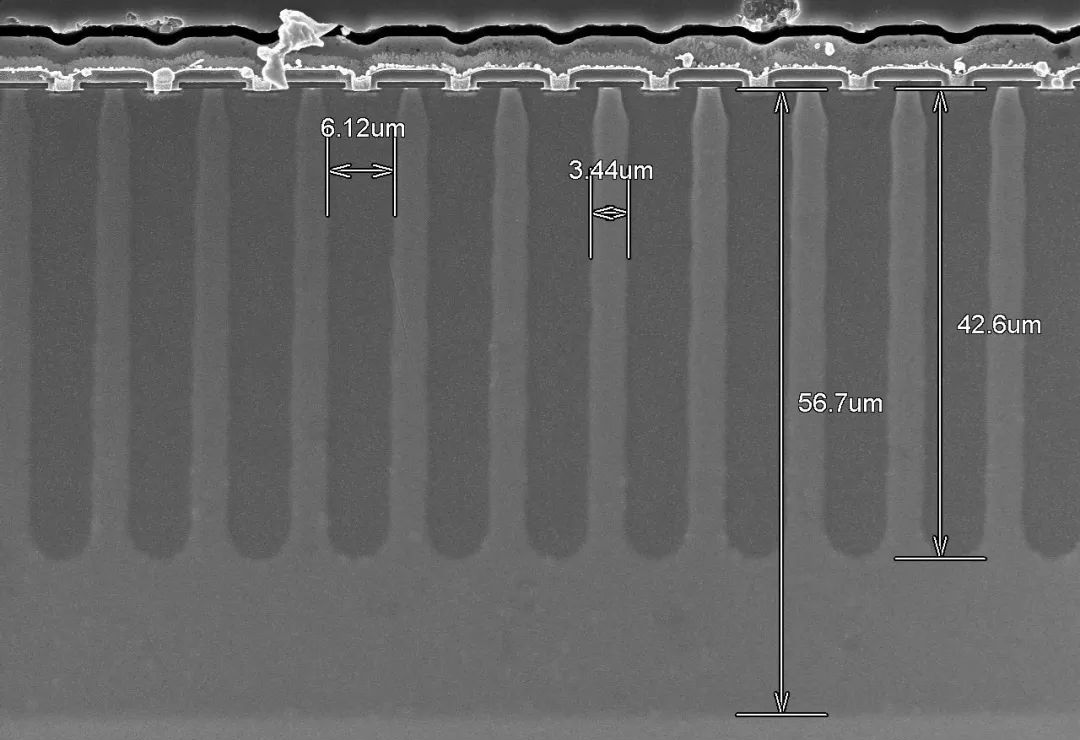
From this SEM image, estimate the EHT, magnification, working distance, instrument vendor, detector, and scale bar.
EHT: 10 kV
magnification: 1.3 K X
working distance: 7.9 mm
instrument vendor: Hitachi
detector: SE(UL)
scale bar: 40000 nm